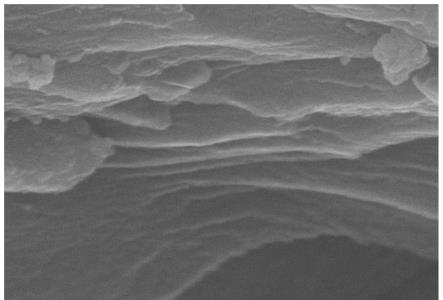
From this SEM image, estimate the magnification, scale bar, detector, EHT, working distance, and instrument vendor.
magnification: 100 K X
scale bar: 500 nm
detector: SE(U)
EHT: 15 kV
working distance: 9.6 mm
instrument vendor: Hitachi